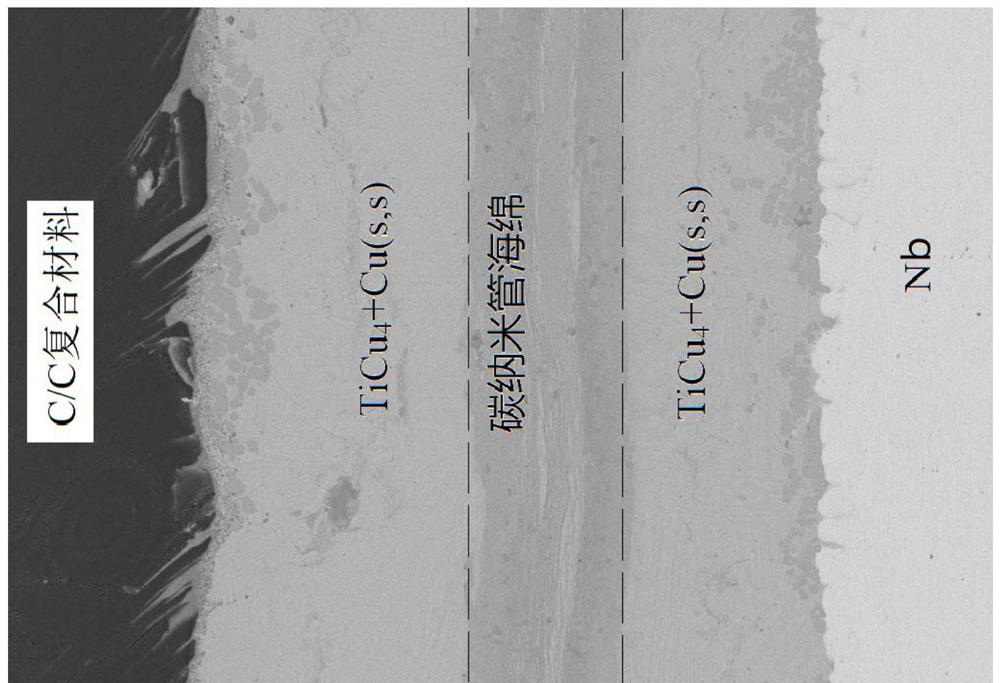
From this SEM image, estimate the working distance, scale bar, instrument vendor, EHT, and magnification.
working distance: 6.3 mm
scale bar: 50000 nm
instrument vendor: FEI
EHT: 20 kV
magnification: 1 K X